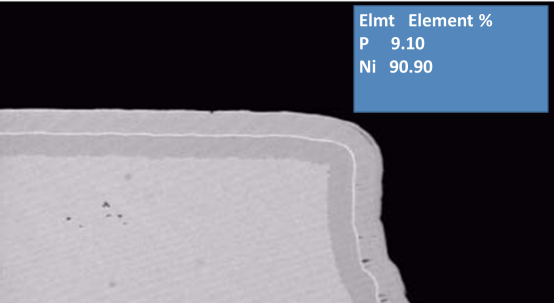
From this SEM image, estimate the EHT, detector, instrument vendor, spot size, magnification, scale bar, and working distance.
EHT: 20 kV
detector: BSE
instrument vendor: FEI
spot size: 5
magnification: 2 K X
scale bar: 20000 nm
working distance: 10 mm